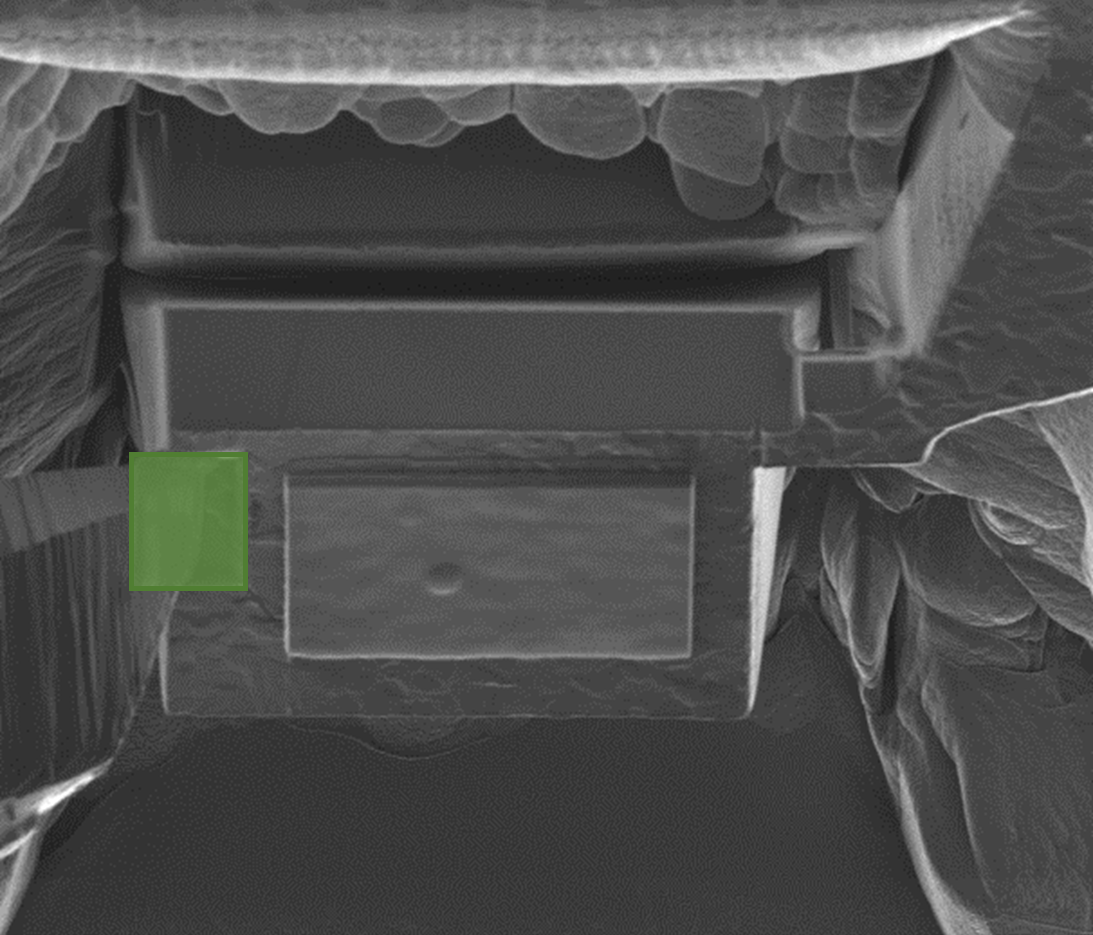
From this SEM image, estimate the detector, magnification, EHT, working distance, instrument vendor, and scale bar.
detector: InLens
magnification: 1.66 K X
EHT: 30 kV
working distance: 5.15 mm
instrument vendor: Zeiss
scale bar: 2000 nm